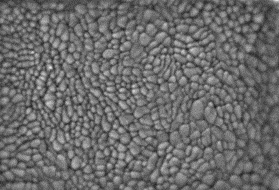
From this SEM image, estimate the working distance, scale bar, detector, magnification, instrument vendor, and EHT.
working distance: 2.1 mm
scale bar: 300 nm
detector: SE+BSE(U)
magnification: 150 K X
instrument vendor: Hitachi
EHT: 1 kV